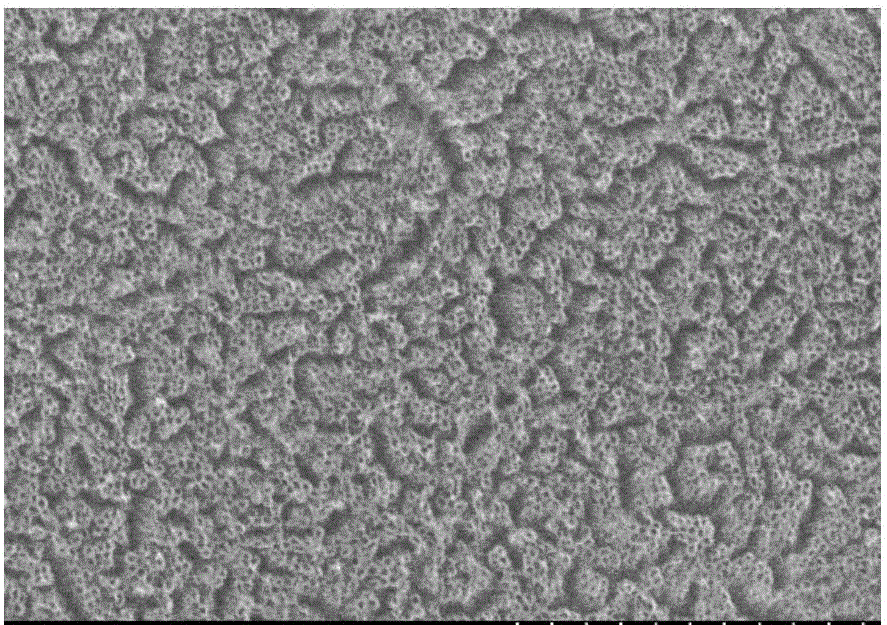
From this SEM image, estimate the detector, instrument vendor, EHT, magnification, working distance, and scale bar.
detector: SE(M)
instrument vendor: Hitachi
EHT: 15 kV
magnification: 50 K X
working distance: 12.1 mm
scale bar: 1000 nm